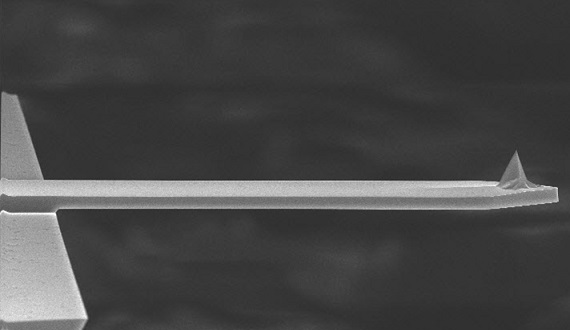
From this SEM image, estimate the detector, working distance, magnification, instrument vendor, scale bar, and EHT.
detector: TLD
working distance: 4.8 mm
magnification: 0.8 K X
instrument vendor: FEI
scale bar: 20000 nm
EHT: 15 kV